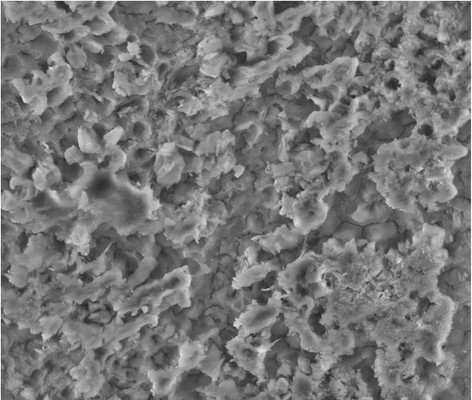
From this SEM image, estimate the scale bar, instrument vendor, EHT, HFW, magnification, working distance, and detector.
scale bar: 50000 nm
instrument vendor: FEI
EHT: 20 kV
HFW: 149 µm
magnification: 2 K X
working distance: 9.9 mm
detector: LFD